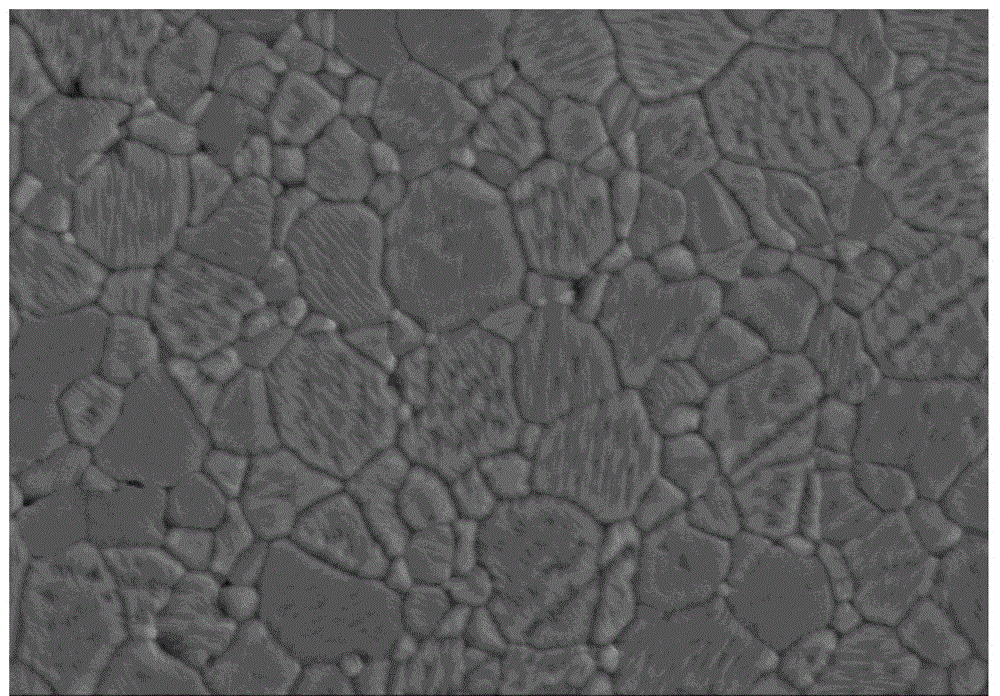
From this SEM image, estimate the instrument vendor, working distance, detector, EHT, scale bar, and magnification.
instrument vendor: Hitachi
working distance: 19.2 mm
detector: SE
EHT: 15 kV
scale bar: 5000 nm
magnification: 6 K X